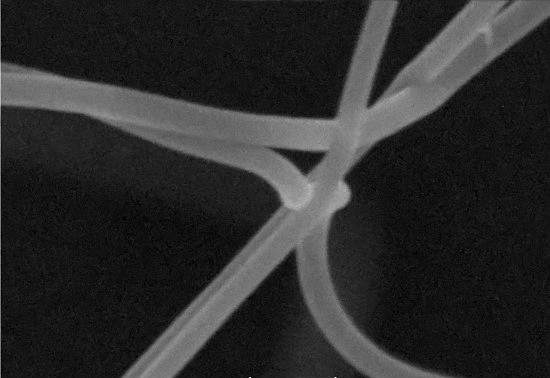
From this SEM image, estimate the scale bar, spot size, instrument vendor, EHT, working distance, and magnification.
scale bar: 300 nm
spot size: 7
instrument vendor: other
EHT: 20 kV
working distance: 5 mm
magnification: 100 K X